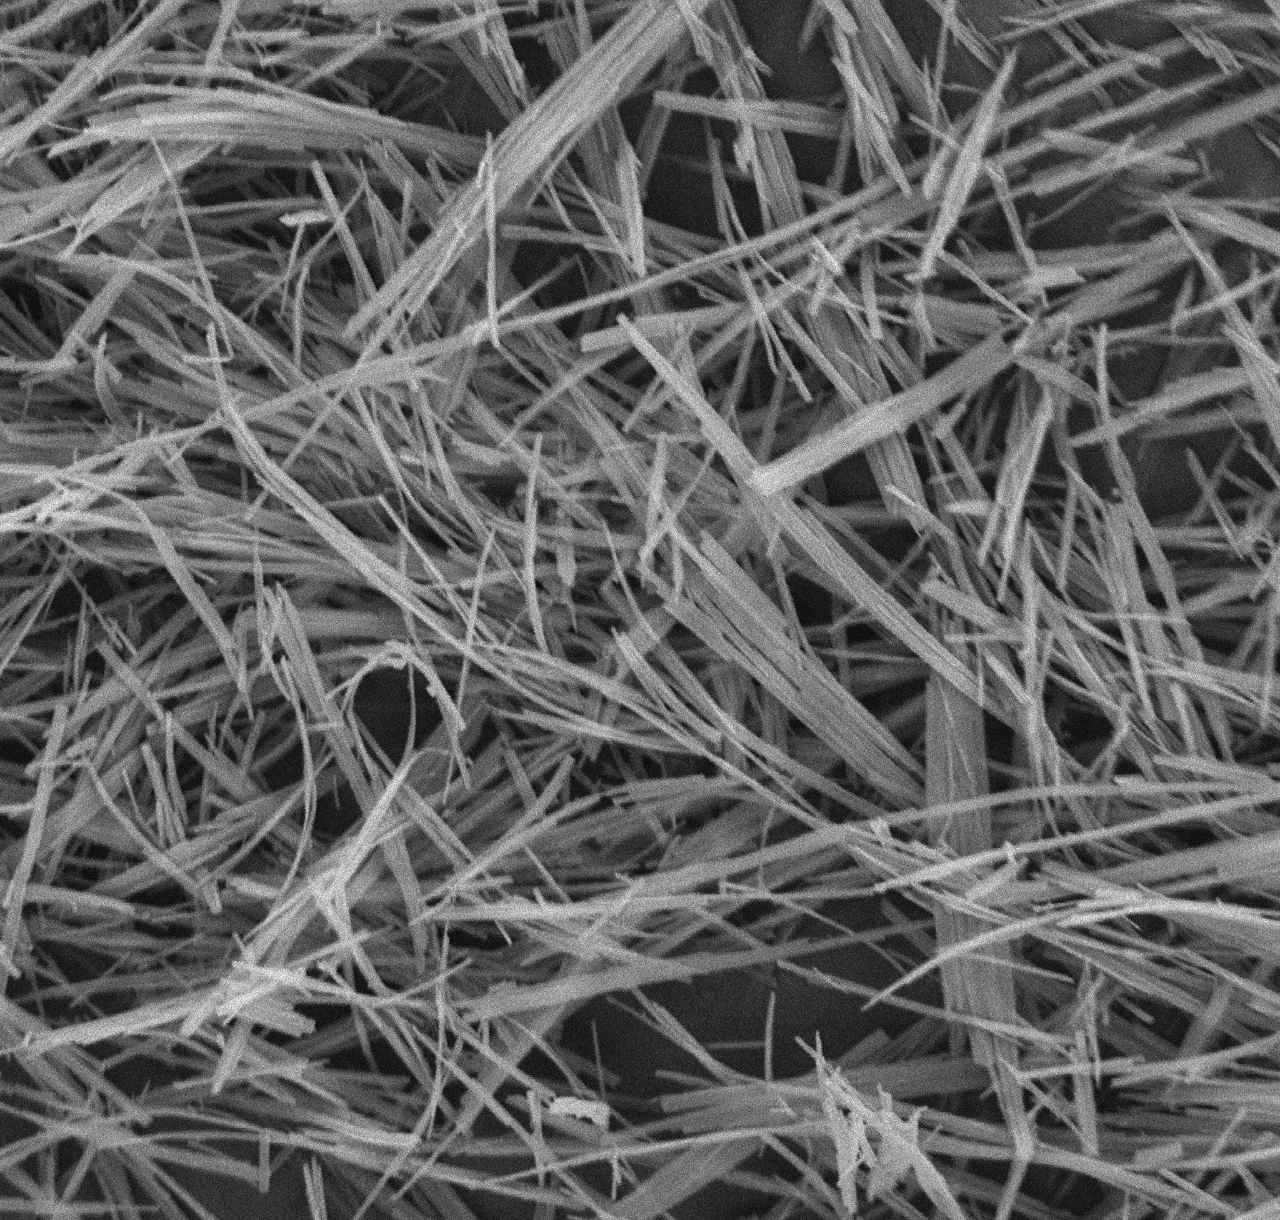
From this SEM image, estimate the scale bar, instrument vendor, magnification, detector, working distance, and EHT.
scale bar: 5000 nm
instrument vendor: other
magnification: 10 K X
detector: BSE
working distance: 9.1 mm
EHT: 15 kV